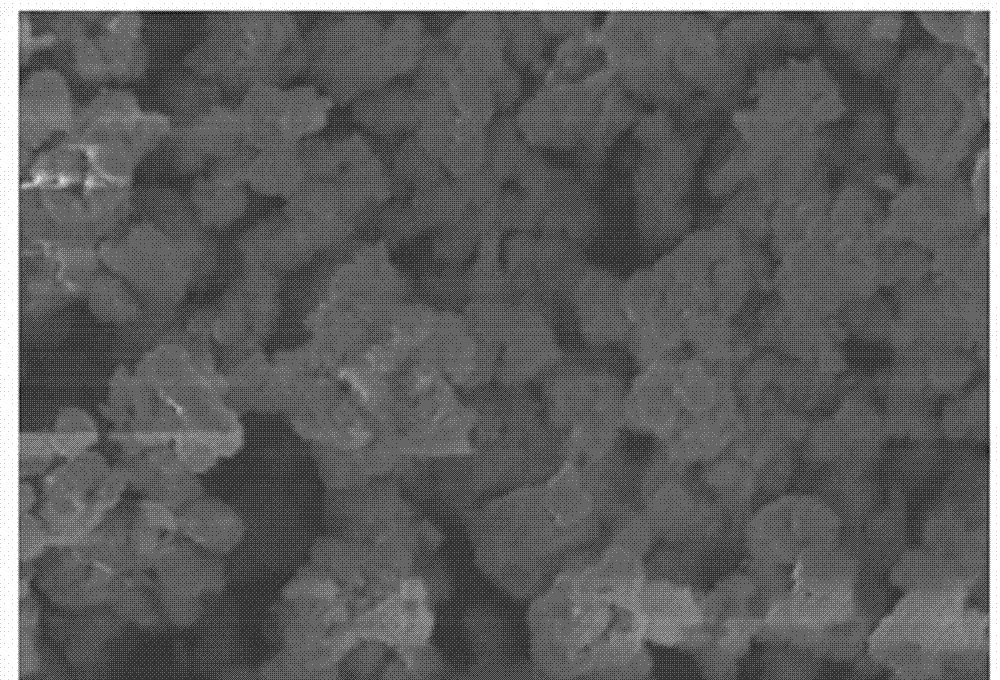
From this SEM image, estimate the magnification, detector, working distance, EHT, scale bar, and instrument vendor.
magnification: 12 K X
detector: SE(UL)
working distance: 4.2 mm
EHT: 5 kV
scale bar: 4000 nm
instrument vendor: Hitachi